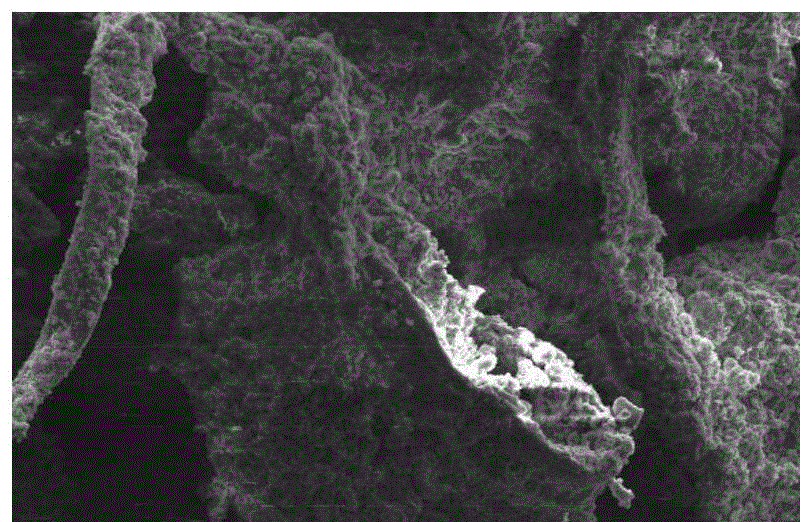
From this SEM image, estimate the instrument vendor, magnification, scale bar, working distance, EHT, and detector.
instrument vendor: Hitachi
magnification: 0.3 K X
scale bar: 100000 nm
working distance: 13.8 mm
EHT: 15 kV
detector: SE(M)